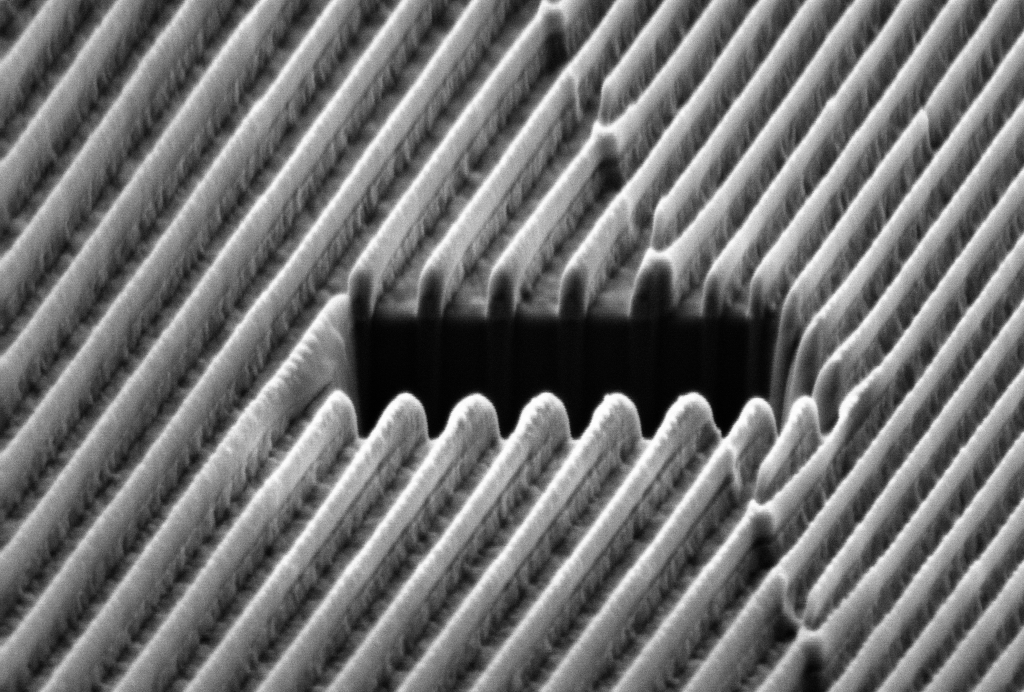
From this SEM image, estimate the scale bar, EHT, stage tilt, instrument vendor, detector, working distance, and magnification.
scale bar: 200 nm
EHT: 2 kV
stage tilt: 54°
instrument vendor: Zeiss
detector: SESI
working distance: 5 mm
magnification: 22.89 K X